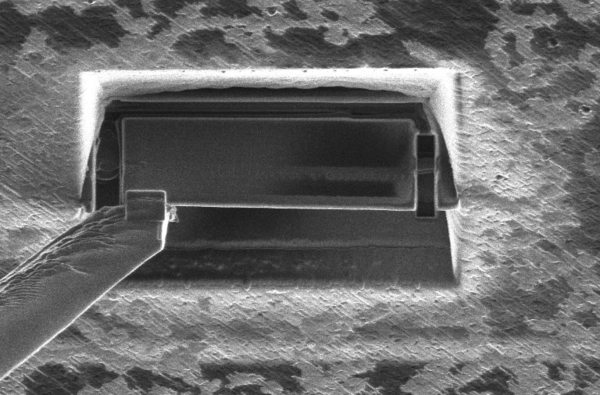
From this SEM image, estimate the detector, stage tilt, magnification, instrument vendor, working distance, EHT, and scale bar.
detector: ETD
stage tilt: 0°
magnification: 6.5 K X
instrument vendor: Thermo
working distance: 19 mm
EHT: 30 kV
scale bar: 5000 nm